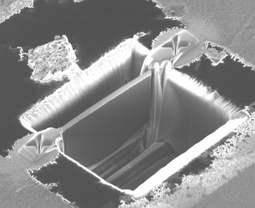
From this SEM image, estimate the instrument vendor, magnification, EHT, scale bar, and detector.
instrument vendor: FEI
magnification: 10 K X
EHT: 30 kV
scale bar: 5000 nm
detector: CDM-E